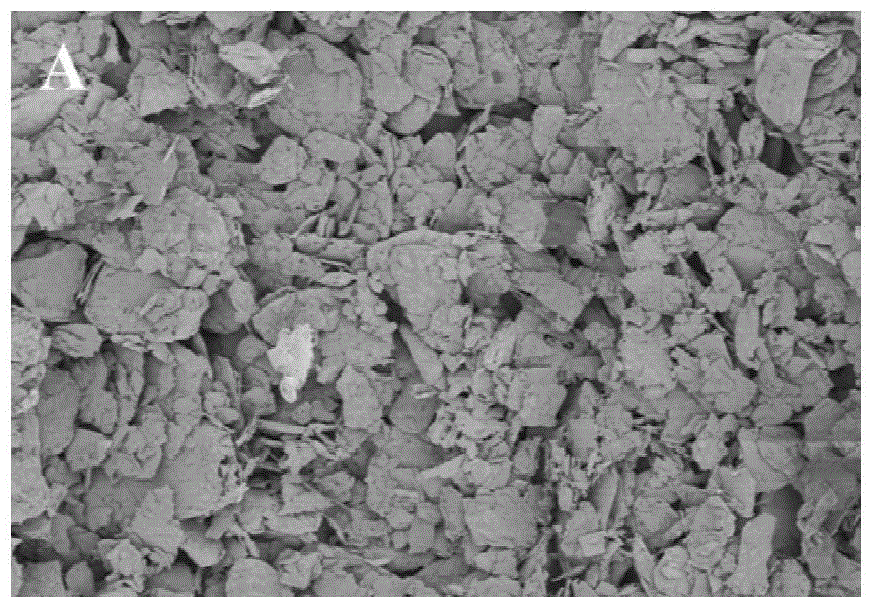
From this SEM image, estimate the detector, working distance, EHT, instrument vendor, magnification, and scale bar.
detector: SE(UL)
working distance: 9.2 mm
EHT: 1 kV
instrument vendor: Hitachi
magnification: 0.5 K X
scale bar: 100000 nm